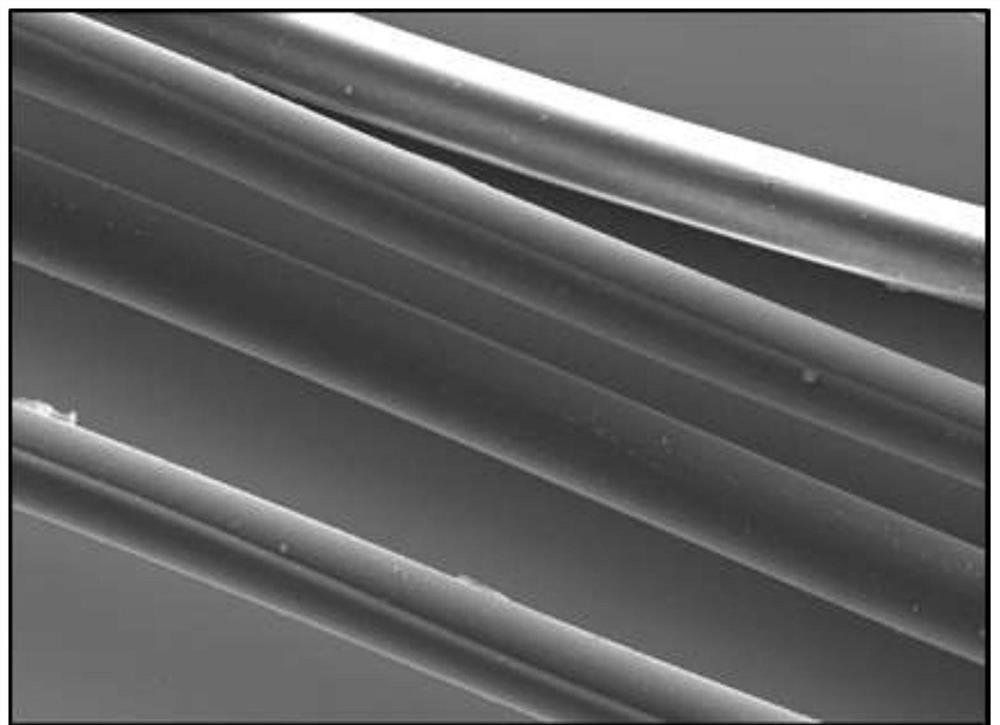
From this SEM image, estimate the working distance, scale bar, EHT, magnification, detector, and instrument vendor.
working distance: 7.9 mm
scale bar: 20000 nm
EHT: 20 kV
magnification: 0.3 K X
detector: SE2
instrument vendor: Zeiss